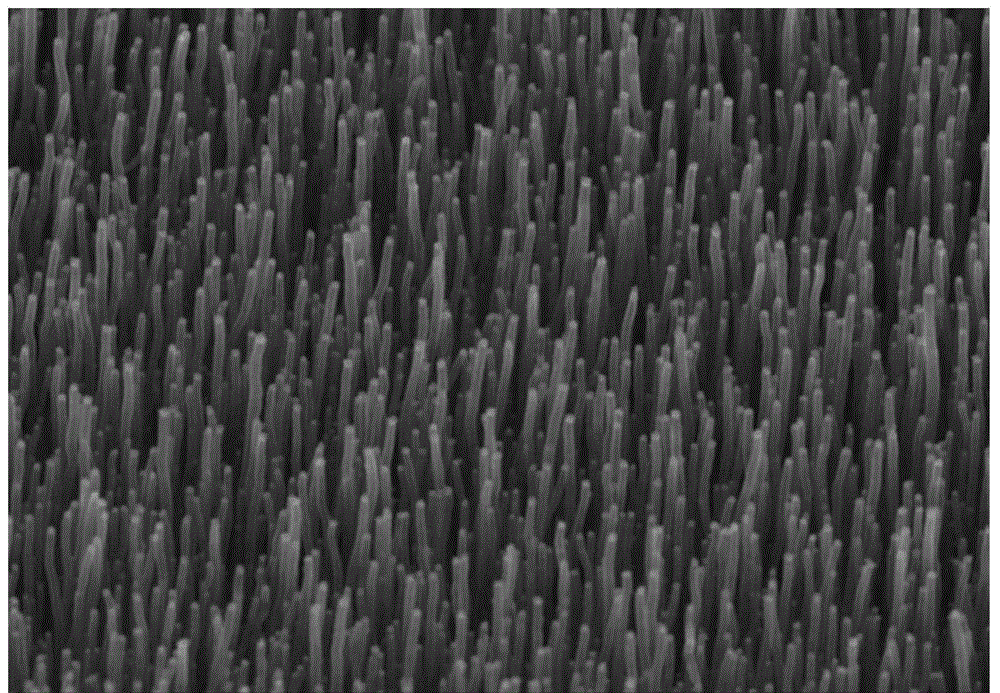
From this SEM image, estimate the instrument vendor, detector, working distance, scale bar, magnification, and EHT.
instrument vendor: Hitachi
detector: SE(M)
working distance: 8.2 mm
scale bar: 2000 nm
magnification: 20 K X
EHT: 15 kV